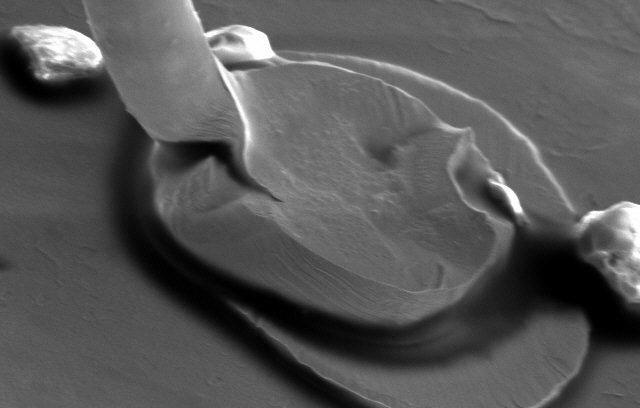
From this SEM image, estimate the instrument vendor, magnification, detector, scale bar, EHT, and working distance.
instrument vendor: JEOL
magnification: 0.8 K X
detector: SE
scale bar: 50000 nm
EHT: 20 kV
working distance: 43.8 mm